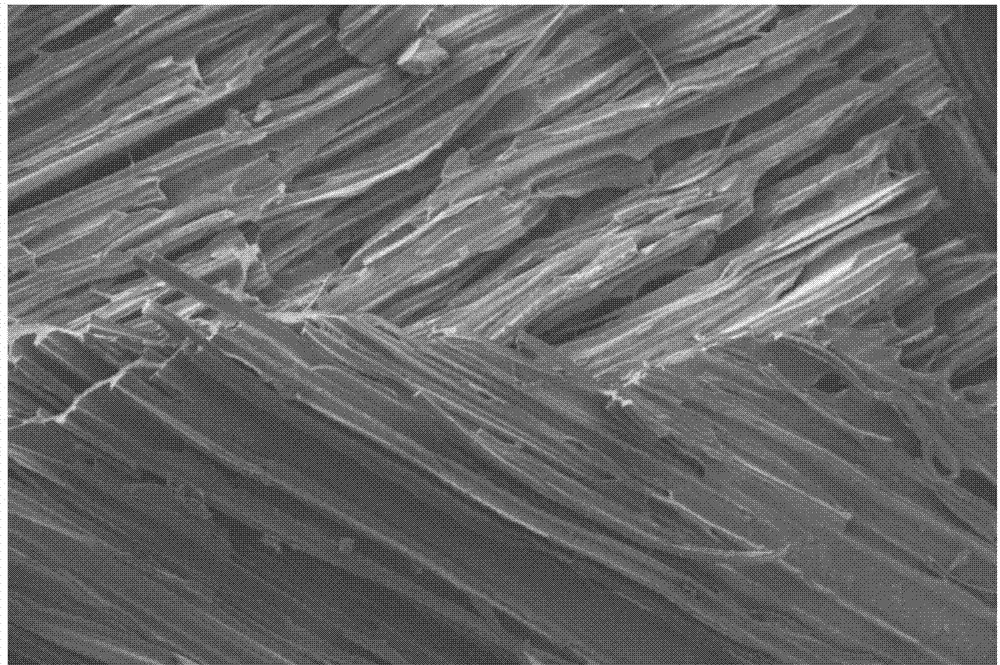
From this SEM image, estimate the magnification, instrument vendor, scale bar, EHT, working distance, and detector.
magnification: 0.025 K X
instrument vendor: Zeiss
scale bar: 200000 nm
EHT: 10 kV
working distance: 16.3 mm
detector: SE2